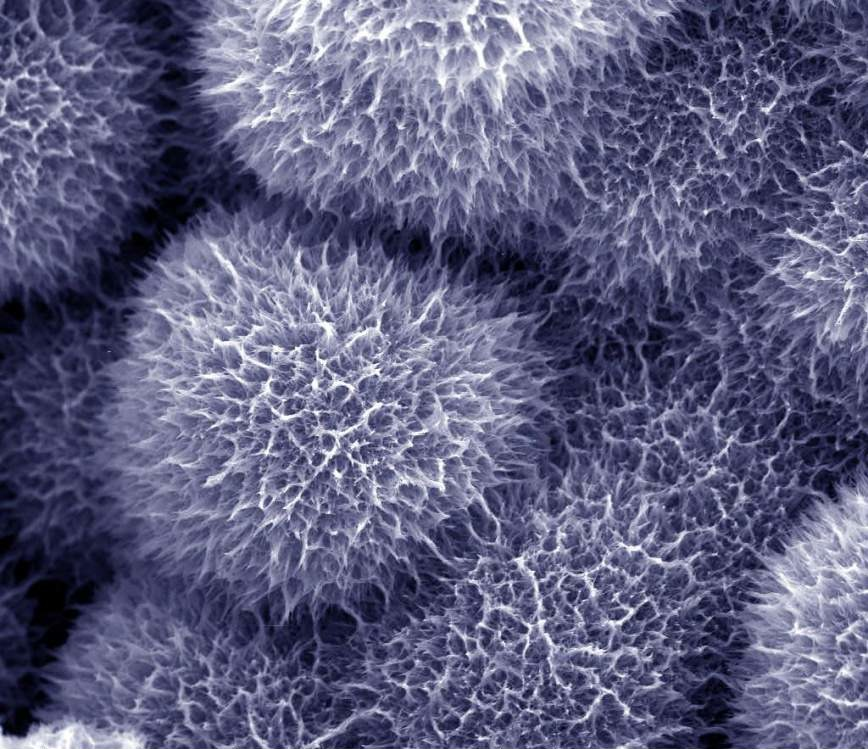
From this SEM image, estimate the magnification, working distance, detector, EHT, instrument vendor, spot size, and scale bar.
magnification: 20 K X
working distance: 4.6 mm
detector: TLD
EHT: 10 kV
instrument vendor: FEI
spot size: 2.5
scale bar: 5000 nm